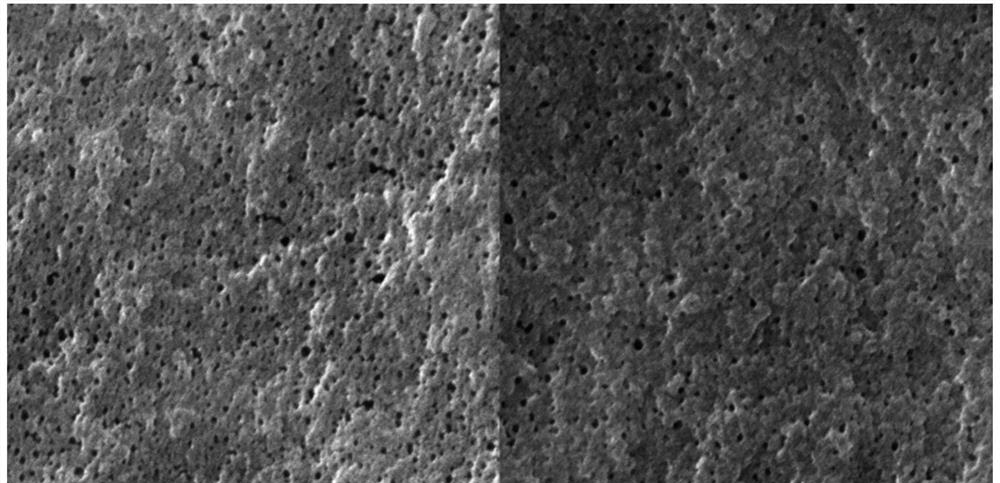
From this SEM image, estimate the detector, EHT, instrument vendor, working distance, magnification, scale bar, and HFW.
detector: SE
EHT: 15 kV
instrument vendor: Tescan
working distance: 8.99 mm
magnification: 200 K X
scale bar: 500 nm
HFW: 2.08 µm